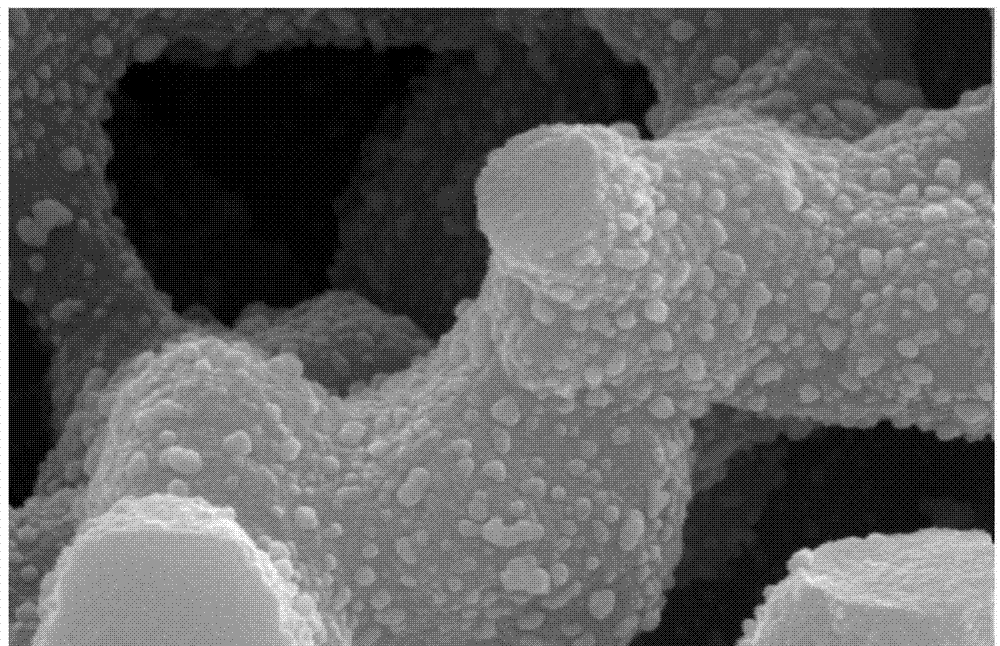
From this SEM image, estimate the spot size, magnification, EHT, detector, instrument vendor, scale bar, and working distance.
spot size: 4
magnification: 50 K X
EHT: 25 kV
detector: SE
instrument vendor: FEI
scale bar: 1000 nm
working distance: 6.2 mm